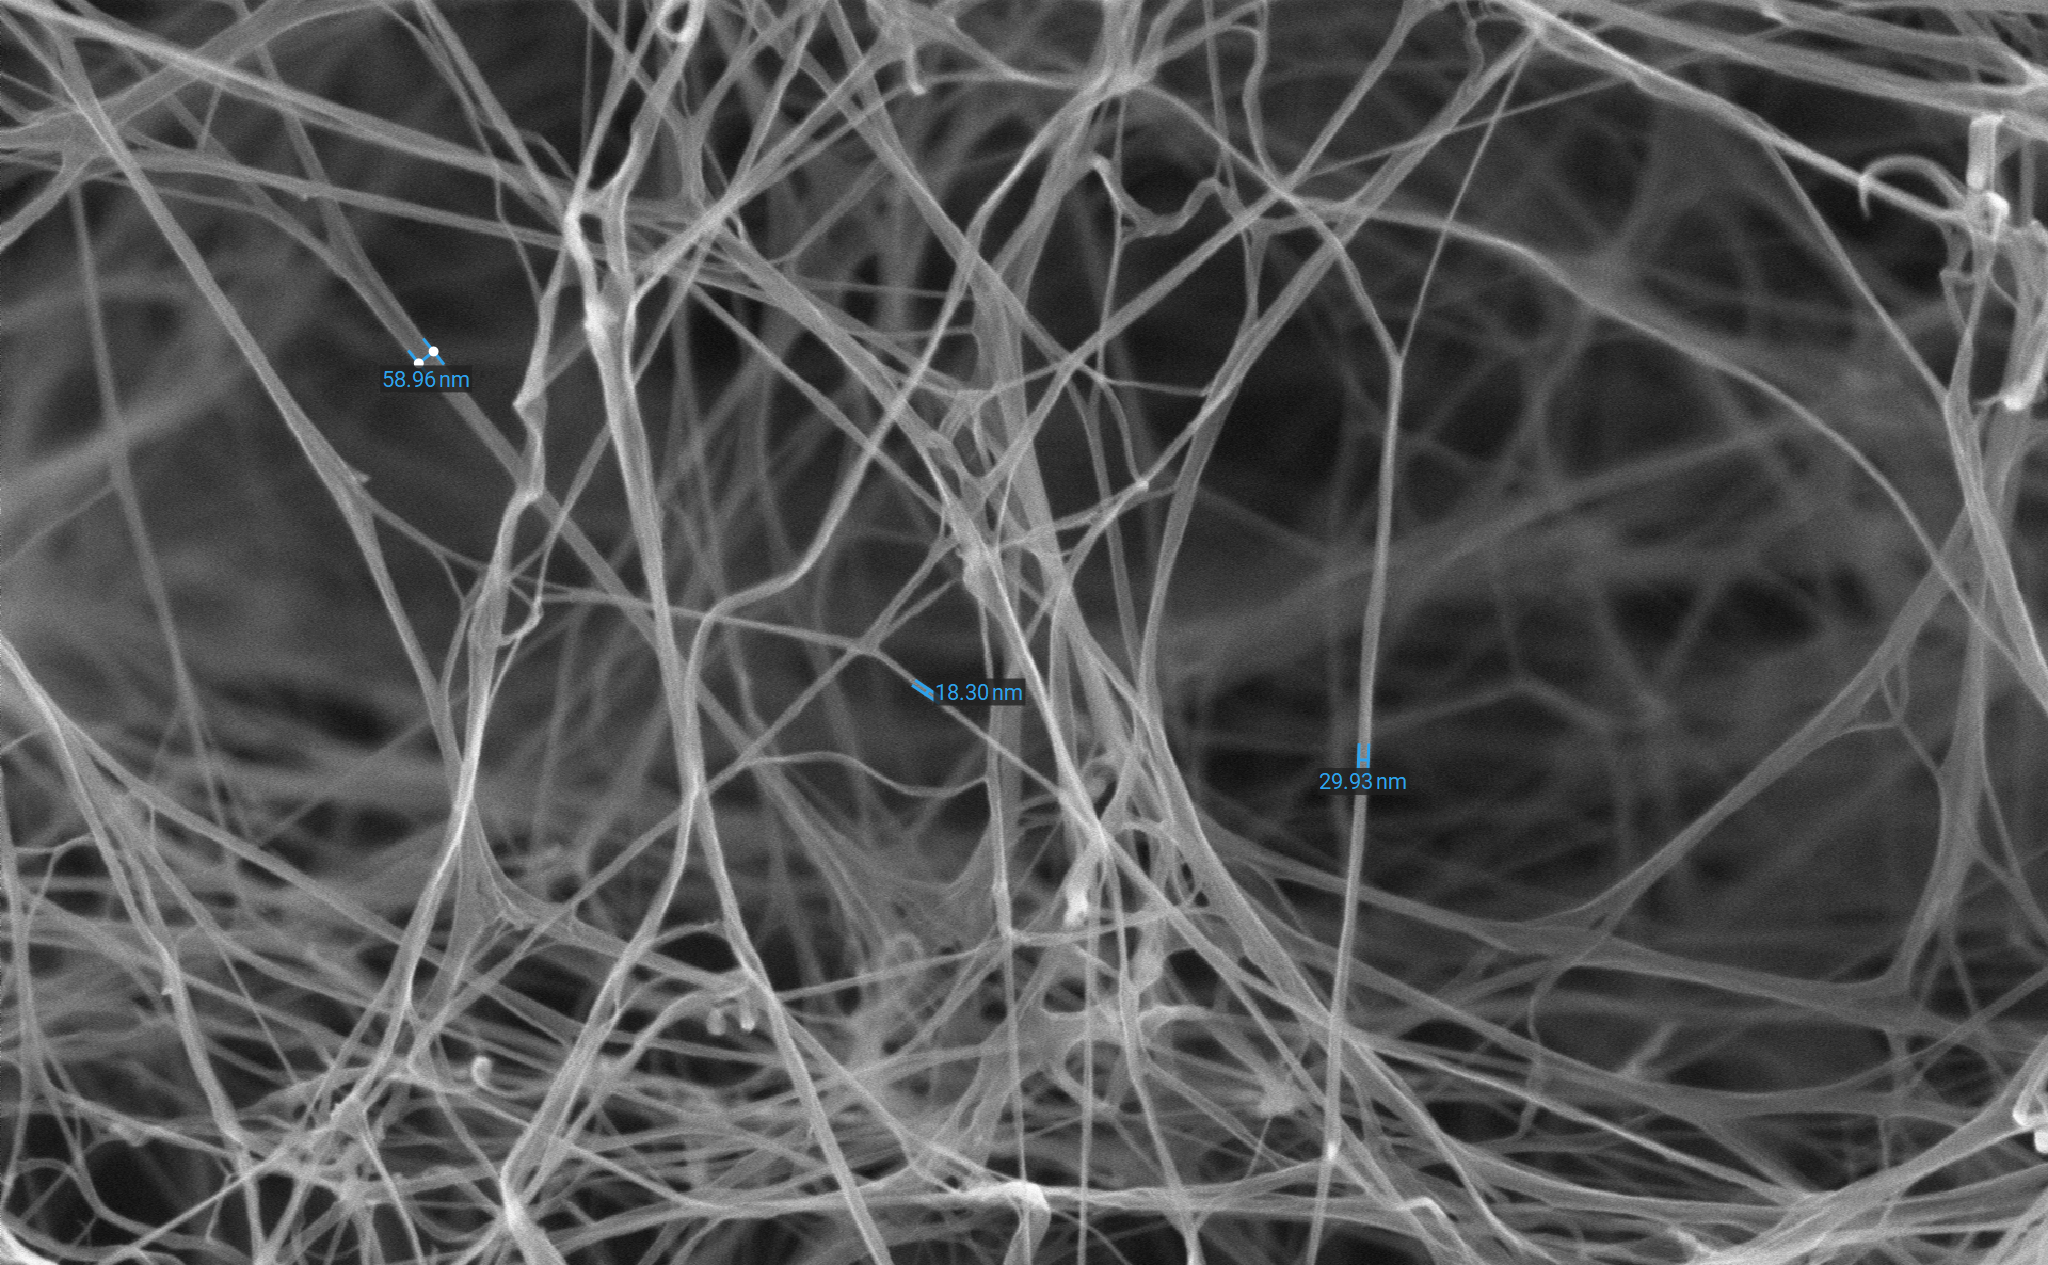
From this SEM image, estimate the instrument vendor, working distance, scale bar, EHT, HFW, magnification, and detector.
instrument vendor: Phenom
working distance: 9.057 mm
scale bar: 2000 nm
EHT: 15 kV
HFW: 6.35 µm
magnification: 20 K X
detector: SED